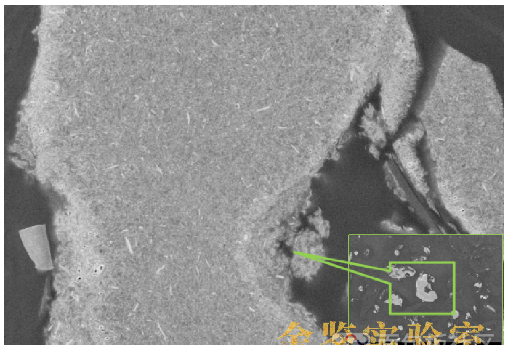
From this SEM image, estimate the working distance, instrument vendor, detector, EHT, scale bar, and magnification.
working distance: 3.3 mm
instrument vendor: Zeiss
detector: InLens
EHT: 5 kV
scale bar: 1000 nm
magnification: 10 K X